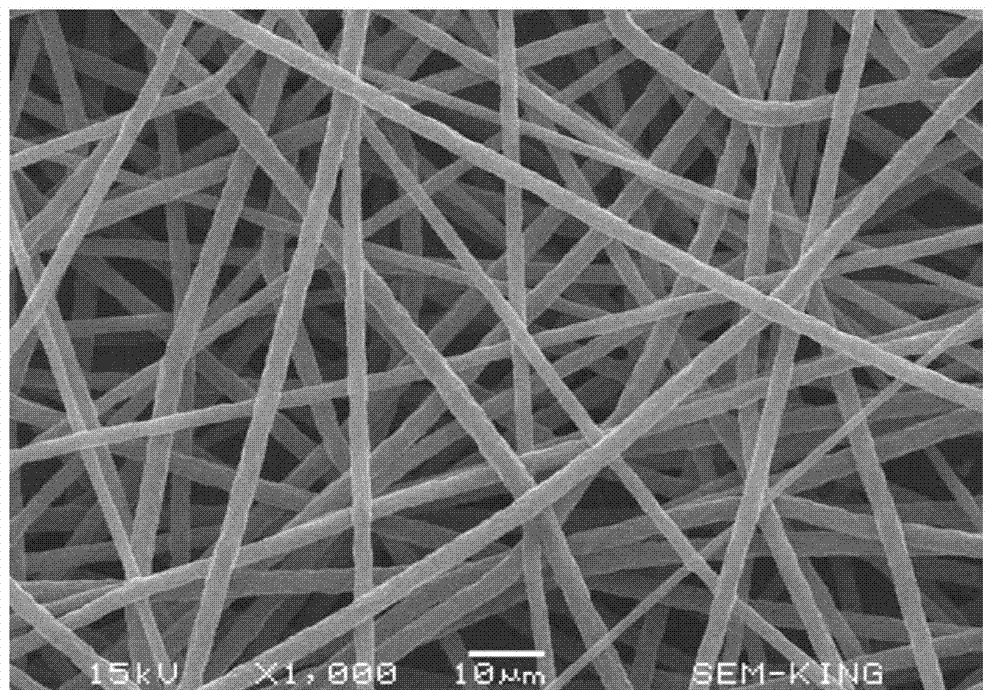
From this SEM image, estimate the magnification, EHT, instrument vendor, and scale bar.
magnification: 1 K X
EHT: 15 kV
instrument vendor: JEOL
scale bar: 10000 nm